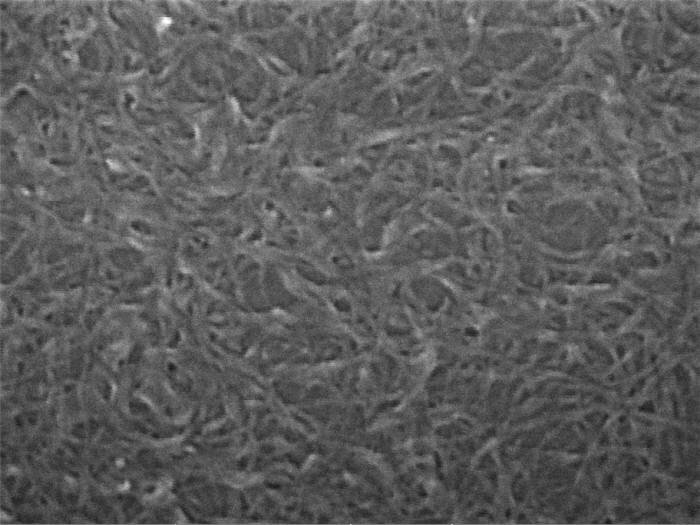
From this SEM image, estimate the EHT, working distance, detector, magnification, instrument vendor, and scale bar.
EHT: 15 kV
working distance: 11.76 mm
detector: InBeam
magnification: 200 K X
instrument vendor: Tescan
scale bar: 200 nm